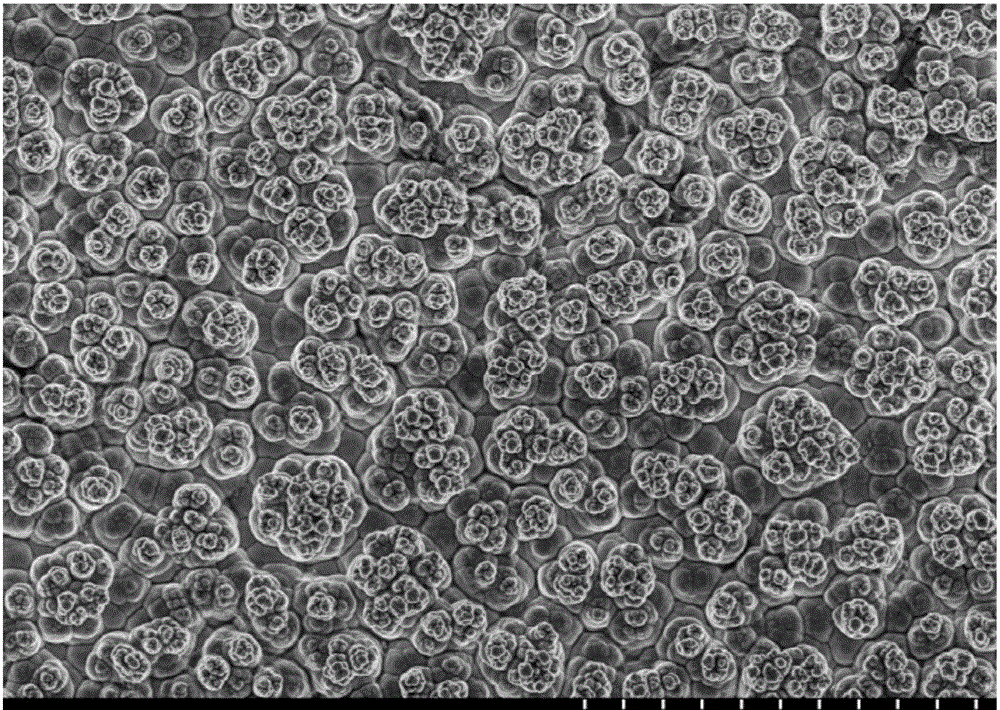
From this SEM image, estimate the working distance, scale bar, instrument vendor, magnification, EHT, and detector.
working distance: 7.9 mm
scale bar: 50000 nm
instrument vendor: Hitachi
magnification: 1 K X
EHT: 10 kV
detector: SE(M)